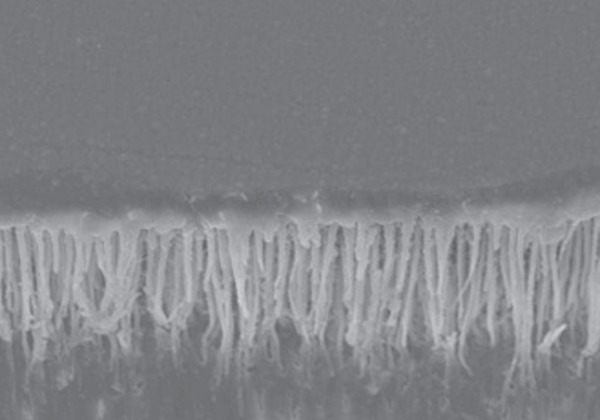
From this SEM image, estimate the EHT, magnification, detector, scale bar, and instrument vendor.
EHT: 25 kV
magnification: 0.5 K X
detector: SEI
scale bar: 50000 nm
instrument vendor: JEOL